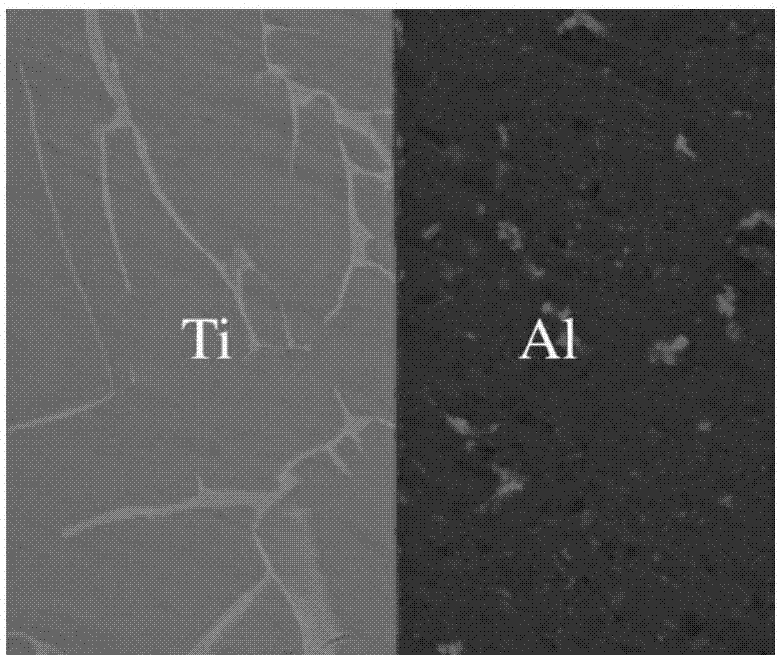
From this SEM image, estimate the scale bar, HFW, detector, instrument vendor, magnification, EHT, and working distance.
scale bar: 20000 nm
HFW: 59.7 µm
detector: BSED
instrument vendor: FEI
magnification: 5 K X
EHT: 20 kV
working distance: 11.6 mm